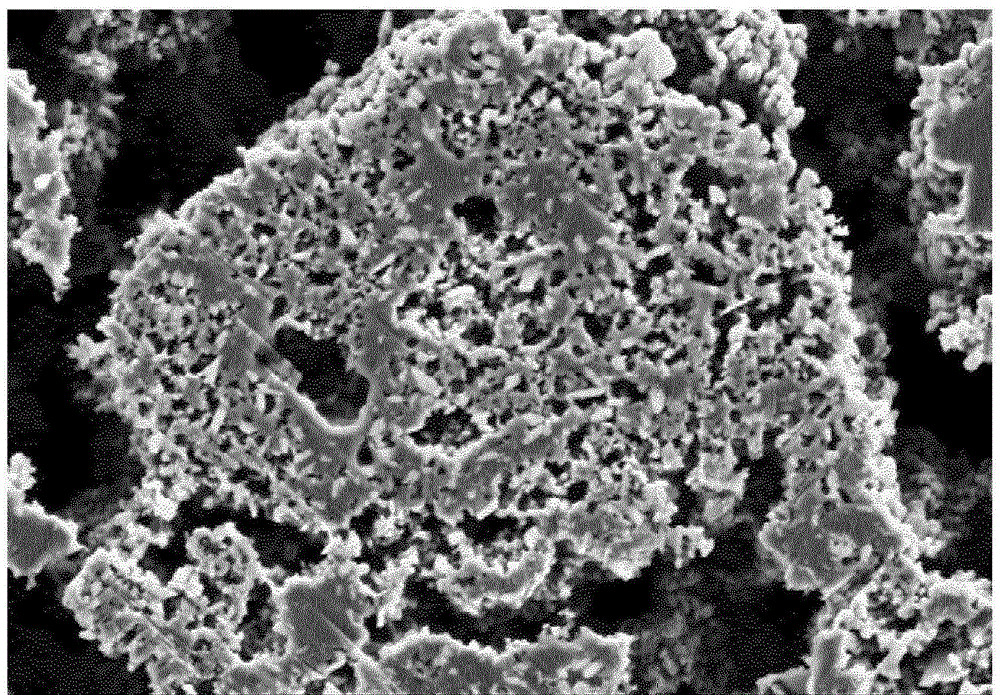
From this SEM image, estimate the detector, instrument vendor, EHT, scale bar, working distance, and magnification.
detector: SE2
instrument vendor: Zeiss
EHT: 3 kV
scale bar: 1000 nm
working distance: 8.9 mm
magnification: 7.5 K X